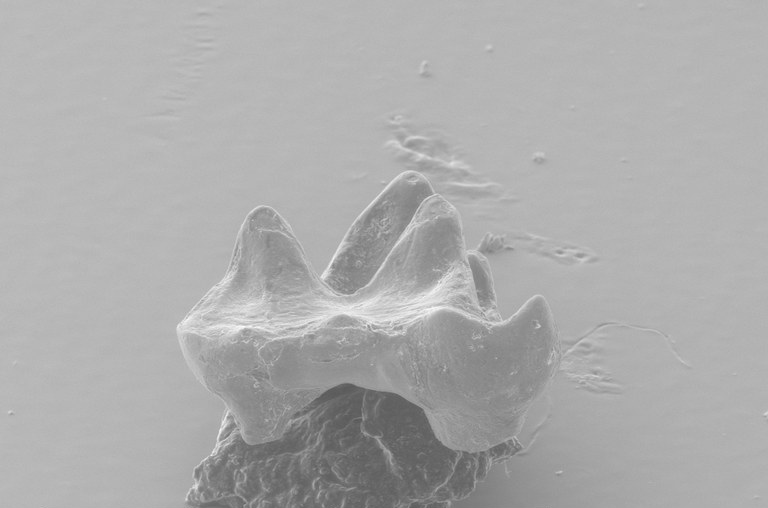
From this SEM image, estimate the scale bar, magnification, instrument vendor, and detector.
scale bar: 500000 nm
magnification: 0.09 K X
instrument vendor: FEI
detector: AUX